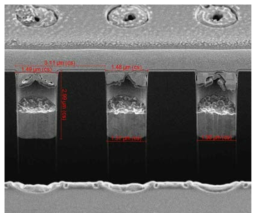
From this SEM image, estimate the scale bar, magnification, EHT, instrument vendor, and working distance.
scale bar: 5000 nm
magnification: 15 K X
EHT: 2 kV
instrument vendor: FEI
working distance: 4.1 mm